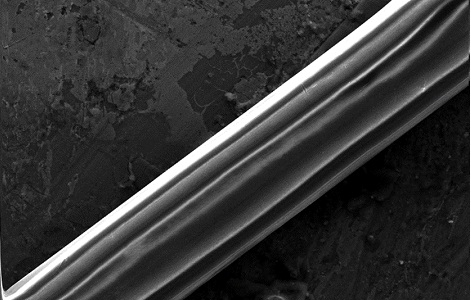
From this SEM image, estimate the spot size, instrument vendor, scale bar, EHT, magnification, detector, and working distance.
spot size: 4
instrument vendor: FEI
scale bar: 50000 nm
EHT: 20 kV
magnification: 1.293 K X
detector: SE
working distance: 6.4 mm